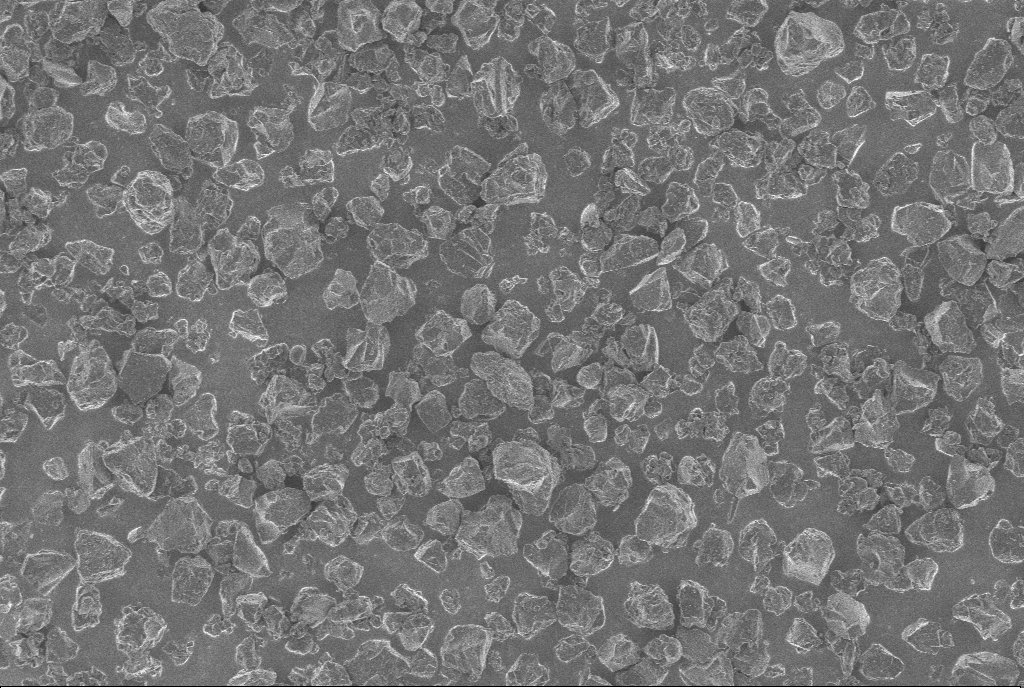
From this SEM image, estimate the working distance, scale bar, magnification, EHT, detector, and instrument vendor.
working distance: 5.6 mm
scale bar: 10000 nm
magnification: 1 K X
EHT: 1 kV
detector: InLens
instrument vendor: Zeiss